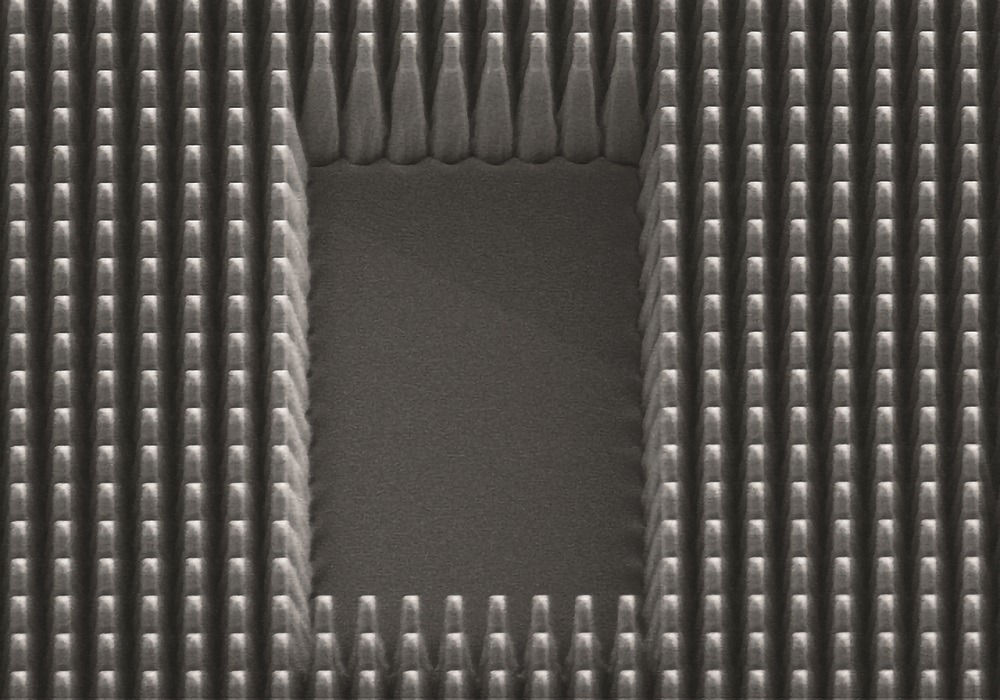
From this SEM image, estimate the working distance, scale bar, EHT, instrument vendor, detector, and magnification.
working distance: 11.7 mm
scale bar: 20000 nm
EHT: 5 kV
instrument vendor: Hitachi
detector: SE(M)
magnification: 2.5 K X